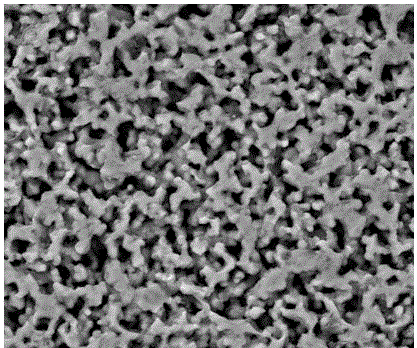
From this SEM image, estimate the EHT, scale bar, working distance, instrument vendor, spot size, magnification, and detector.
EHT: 20 kV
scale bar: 20000 nm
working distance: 10.4 mm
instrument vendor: FEI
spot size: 5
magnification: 2 K X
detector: BSED